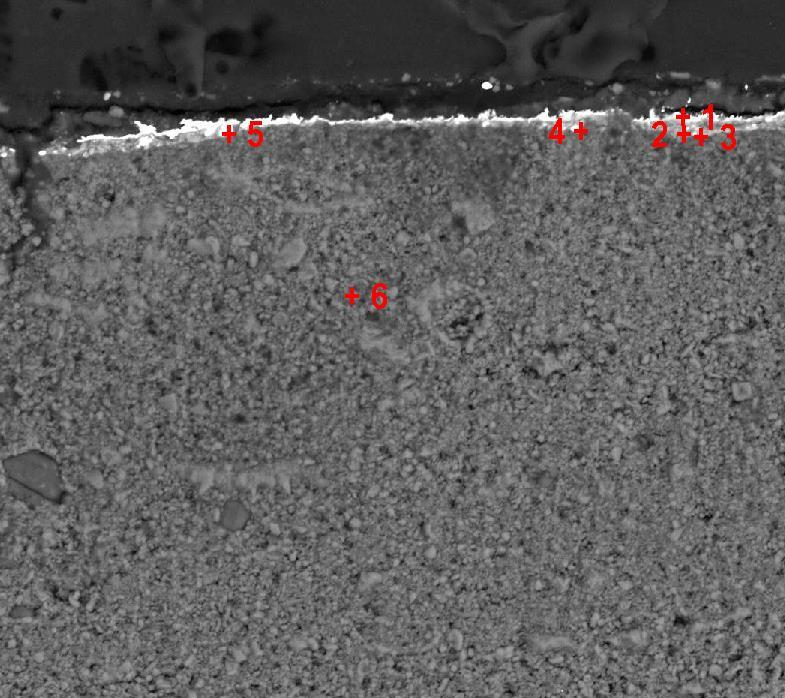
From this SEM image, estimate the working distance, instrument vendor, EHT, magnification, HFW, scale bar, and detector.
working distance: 11.33 mm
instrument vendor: Tescan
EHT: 20 kV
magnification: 1.62 K X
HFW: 196 µm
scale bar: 50000 nm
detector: BSE